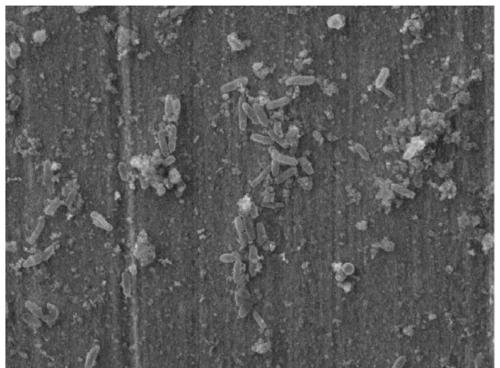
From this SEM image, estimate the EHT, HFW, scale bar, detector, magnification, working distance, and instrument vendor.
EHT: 20 kV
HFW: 39.6 µm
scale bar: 10000 nm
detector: SE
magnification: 6.98 K X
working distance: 17.52 mm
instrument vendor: Tescan